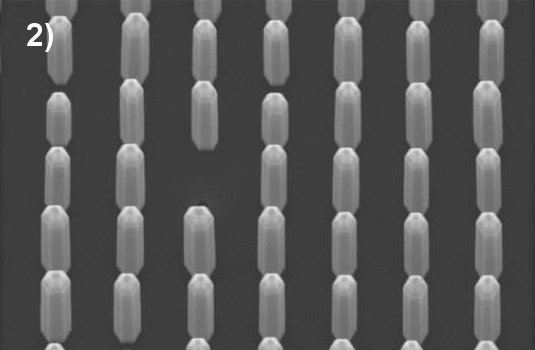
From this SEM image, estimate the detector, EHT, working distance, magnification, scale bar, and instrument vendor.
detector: InLens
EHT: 5 kV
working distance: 5.1 mm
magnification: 30 K X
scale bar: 200 nm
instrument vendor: Zeiss